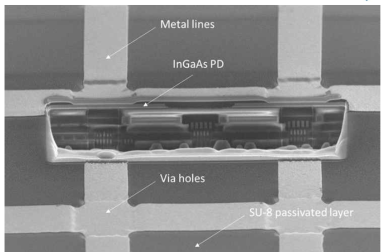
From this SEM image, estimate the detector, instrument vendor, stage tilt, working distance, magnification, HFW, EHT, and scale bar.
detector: TLD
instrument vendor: FEI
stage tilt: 52°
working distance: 4 mm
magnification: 3.5 K X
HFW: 59.2 µm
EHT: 5 kV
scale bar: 10000 nm